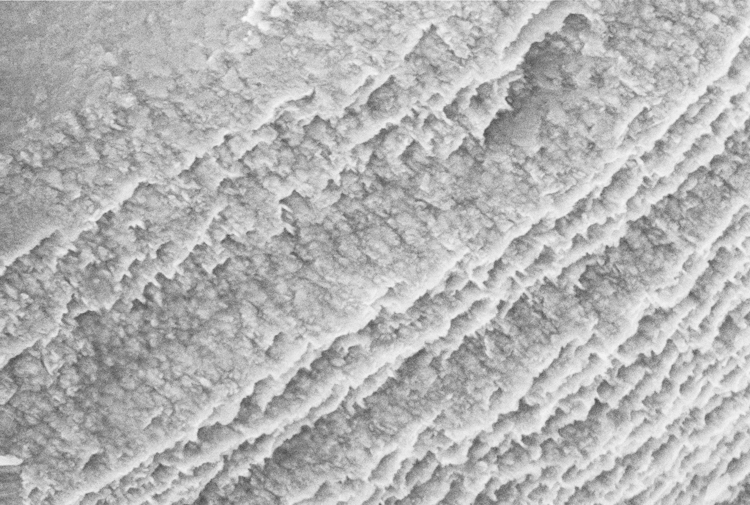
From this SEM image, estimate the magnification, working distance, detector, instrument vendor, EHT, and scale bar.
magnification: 100 K X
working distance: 2.3 mm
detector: InLens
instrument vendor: Zeiss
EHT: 3 kV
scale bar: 200 nm